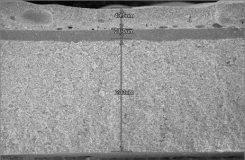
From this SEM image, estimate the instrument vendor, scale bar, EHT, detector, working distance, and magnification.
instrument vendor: Hitachi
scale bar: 200000 nm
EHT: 15 kV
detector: SE(M)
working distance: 13.3 mm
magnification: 0.25 K X